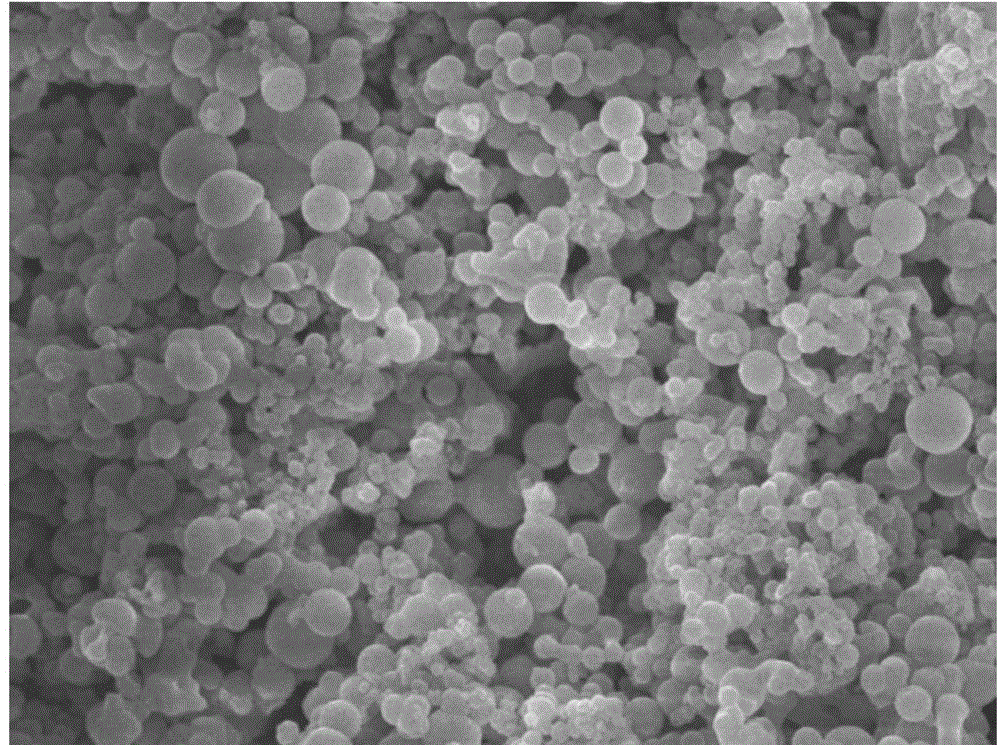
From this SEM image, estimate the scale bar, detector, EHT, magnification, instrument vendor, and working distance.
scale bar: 1000 nm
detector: SEI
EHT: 10 kV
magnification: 7.5 K X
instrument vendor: JEOL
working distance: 7.7 mm